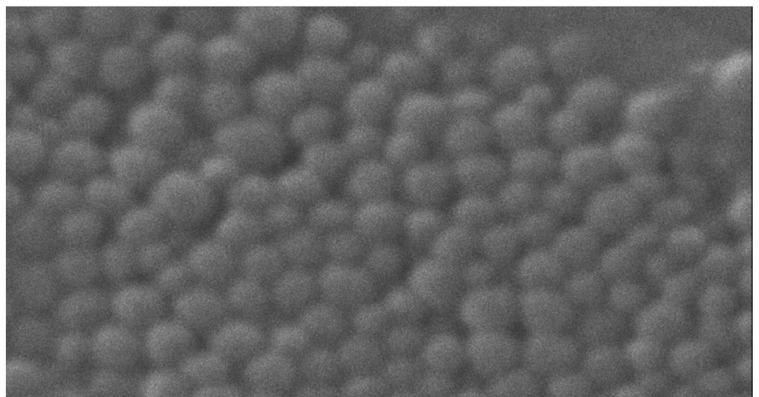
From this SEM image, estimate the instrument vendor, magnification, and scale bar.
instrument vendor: JEOL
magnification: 60 K X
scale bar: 200 nm